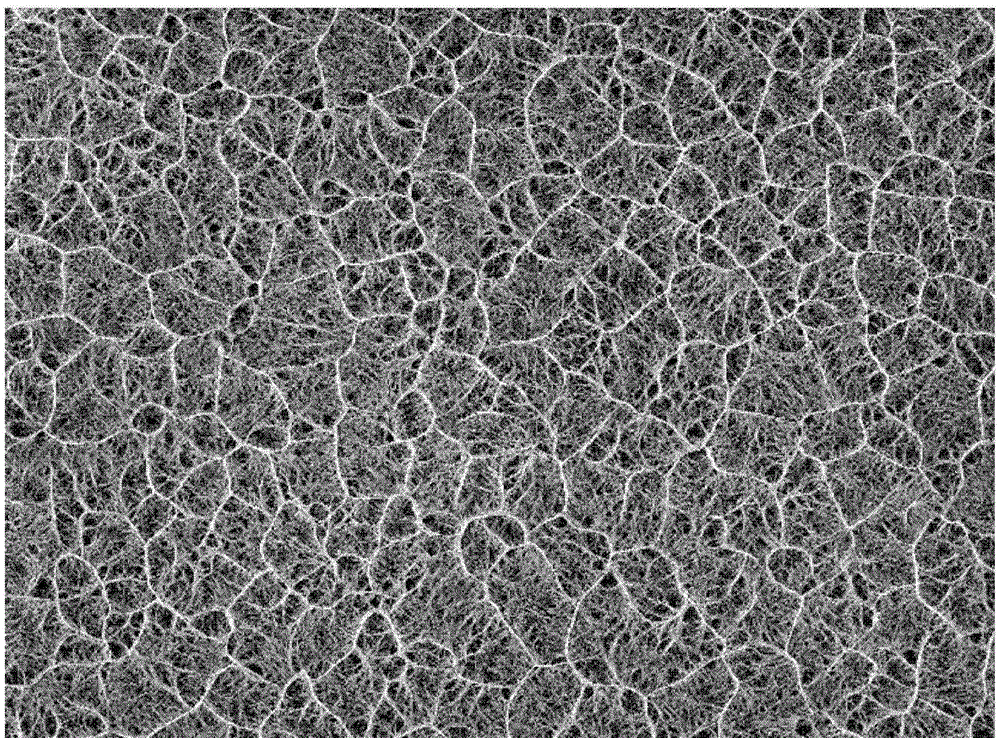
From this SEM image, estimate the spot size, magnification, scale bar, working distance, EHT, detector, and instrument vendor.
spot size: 3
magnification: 0.5 K X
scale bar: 50000 nm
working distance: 6.3 mm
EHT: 10 kV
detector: SE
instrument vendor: FEI